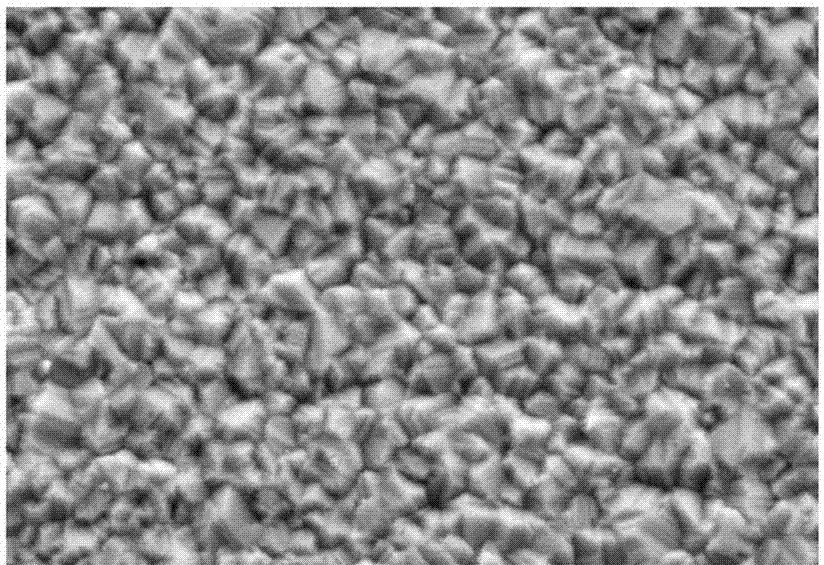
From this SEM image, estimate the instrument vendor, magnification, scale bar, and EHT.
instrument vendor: other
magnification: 3 K X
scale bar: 10000 nm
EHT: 20 kV